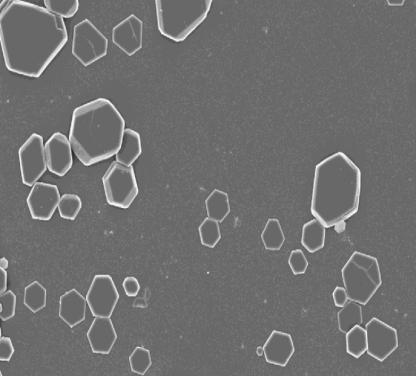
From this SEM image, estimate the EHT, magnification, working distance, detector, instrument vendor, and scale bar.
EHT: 5 kV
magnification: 2 K X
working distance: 6.6 mm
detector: InLens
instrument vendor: Zeiss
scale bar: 10000 nm